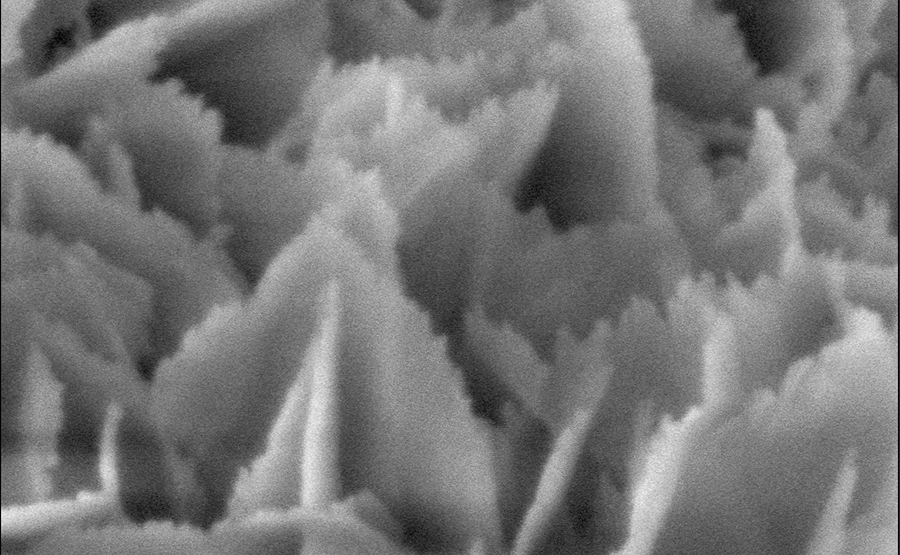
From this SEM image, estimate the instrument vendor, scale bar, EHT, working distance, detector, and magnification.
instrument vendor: Hitachi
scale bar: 100 nm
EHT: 1.5 kV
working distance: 1.1 mm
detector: SE(U)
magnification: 300 K X